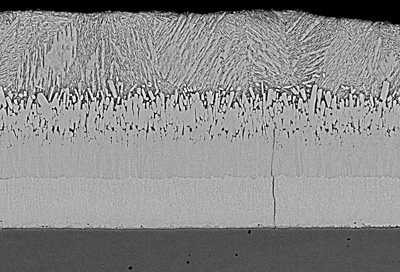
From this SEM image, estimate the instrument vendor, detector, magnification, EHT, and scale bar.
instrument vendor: Hitachi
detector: BSECOMP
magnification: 0.7 K X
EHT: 25 kV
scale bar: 50000 nm